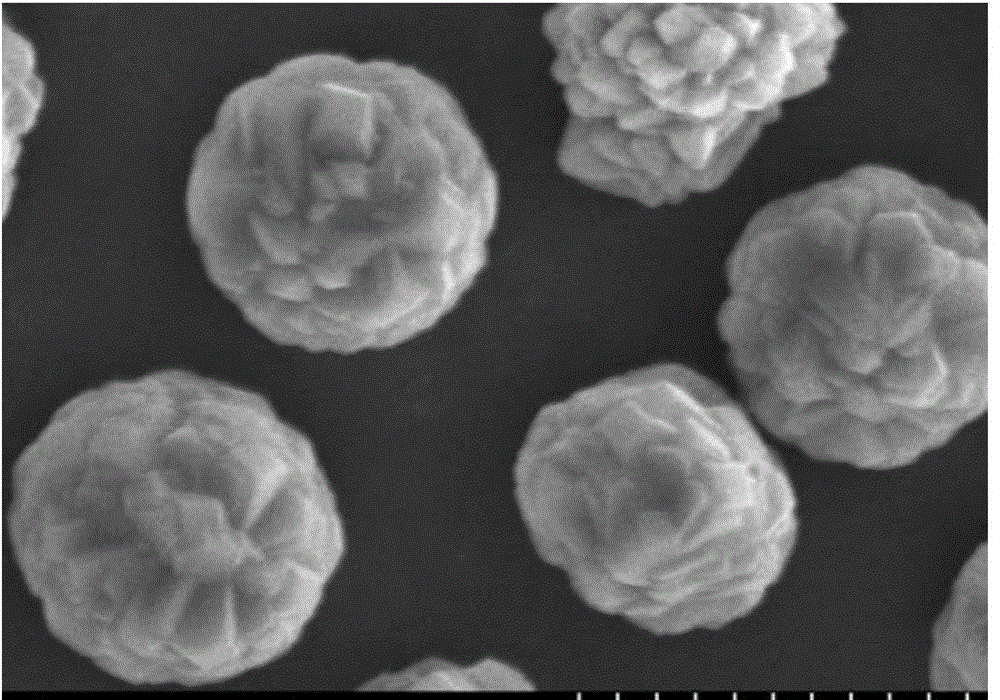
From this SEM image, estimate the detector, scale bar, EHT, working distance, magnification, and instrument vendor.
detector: SE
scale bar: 5000 nm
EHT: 10 kV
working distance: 5.5 mm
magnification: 10 K X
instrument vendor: Hitachi